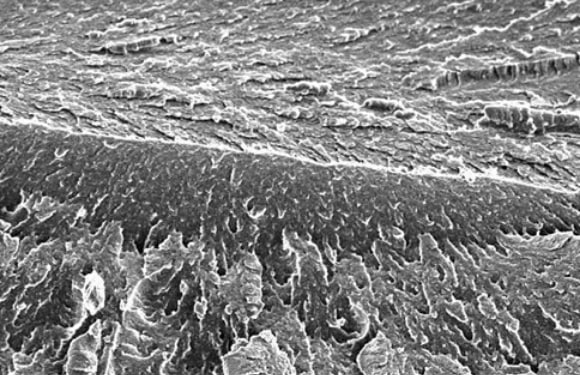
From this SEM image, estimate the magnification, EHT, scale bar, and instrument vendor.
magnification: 0.1 K X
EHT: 20 kV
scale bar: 100000 nm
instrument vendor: JEOL